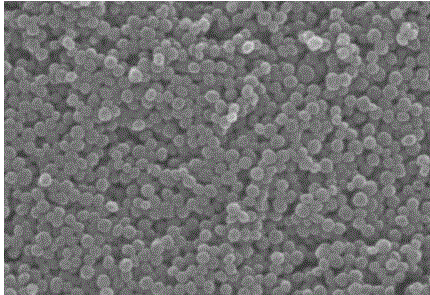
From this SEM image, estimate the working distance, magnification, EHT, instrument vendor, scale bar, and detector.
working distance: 10.9 mm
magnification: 20 K X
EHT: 30 kV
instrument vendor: Hitachi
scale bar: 2000 nm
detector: SE